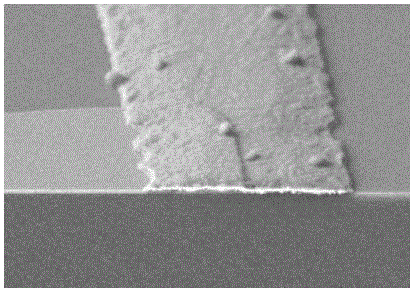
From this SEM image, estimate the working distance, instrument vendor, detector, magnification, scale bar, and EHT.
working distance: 9.1 mm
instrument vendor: Hitachi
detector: SE(M)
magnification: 15 K X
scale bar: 3000 nm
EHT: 5 kV